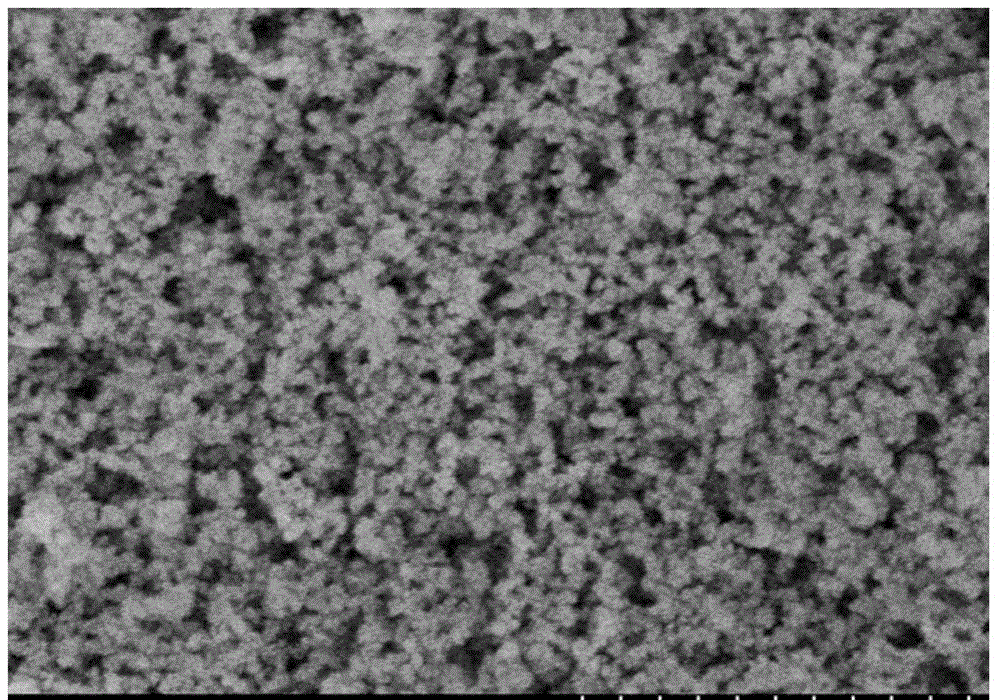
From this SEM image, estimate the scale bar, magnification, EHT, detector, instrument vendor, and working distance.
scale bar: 1000 nm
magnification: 50 K X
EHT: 3 kV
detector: SE(M)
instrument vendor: Hitachi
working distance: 8.6 mm